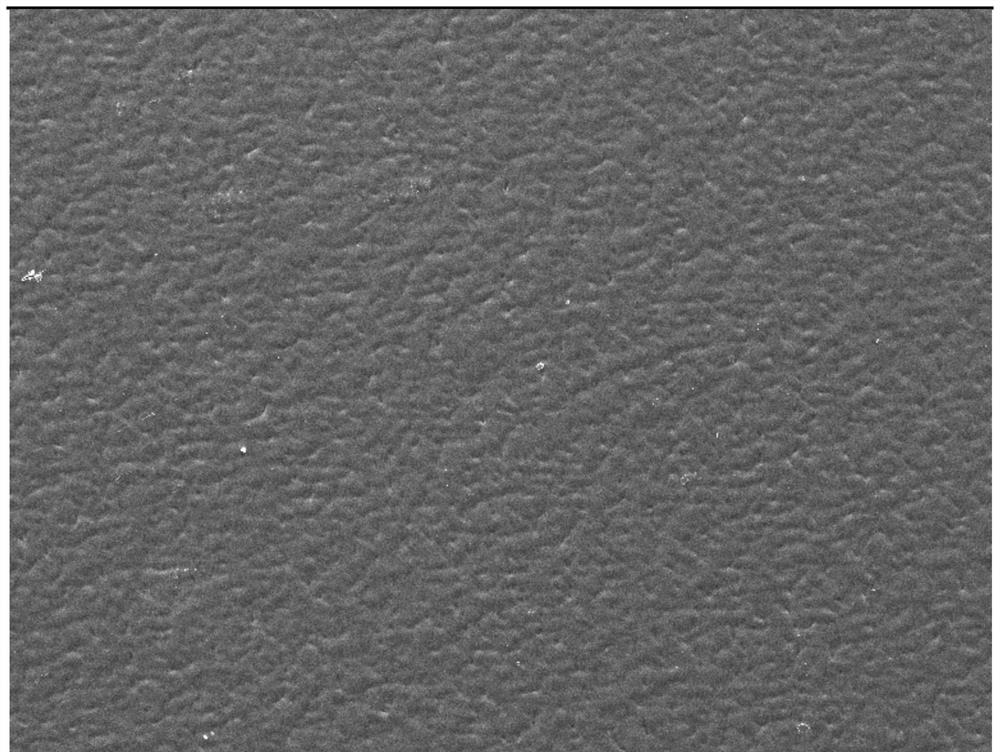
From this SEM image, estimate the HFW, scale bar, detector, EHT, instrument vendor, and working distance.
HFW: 1020 µm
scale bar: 200000 nm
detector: SE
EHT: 30 kV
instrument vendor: Tescan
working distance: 8.51 mm